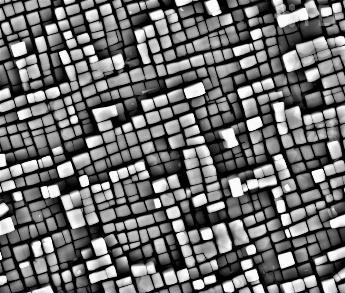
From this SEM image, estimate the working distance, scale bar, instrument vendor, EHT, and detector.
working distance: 7.3 mm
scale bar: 5000 nm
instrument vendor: FEI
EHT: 15 kV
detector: BSED